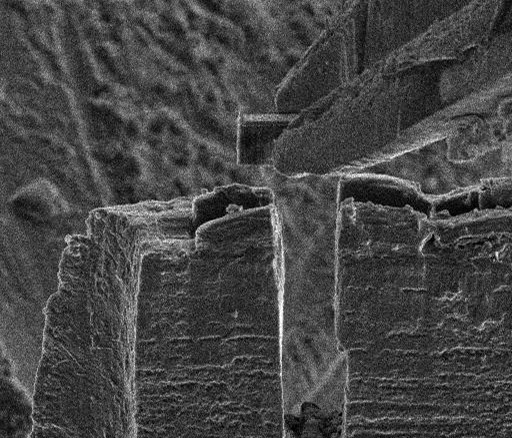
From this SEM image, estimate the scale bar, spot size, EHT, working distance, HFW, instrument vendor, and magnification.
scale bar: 20000 nm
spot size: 4.5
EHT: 5 kV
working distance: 10 mm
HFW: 75.8 µm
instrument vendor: FEI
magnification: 1.5 K X